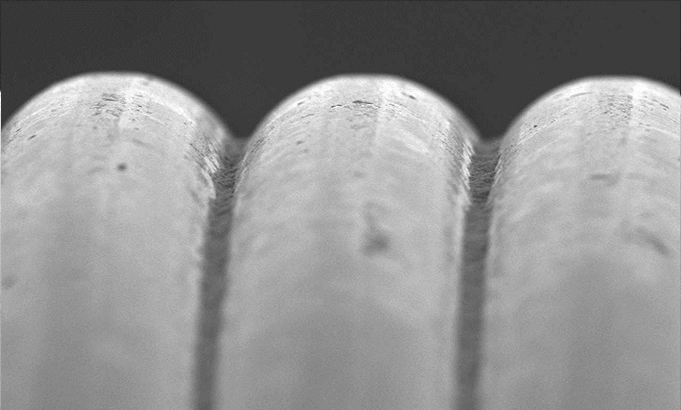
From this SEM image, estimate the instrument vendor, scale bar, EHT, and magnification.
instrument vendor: JEOL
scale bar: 50000 nm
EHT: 10 kV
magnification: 0.5 K X